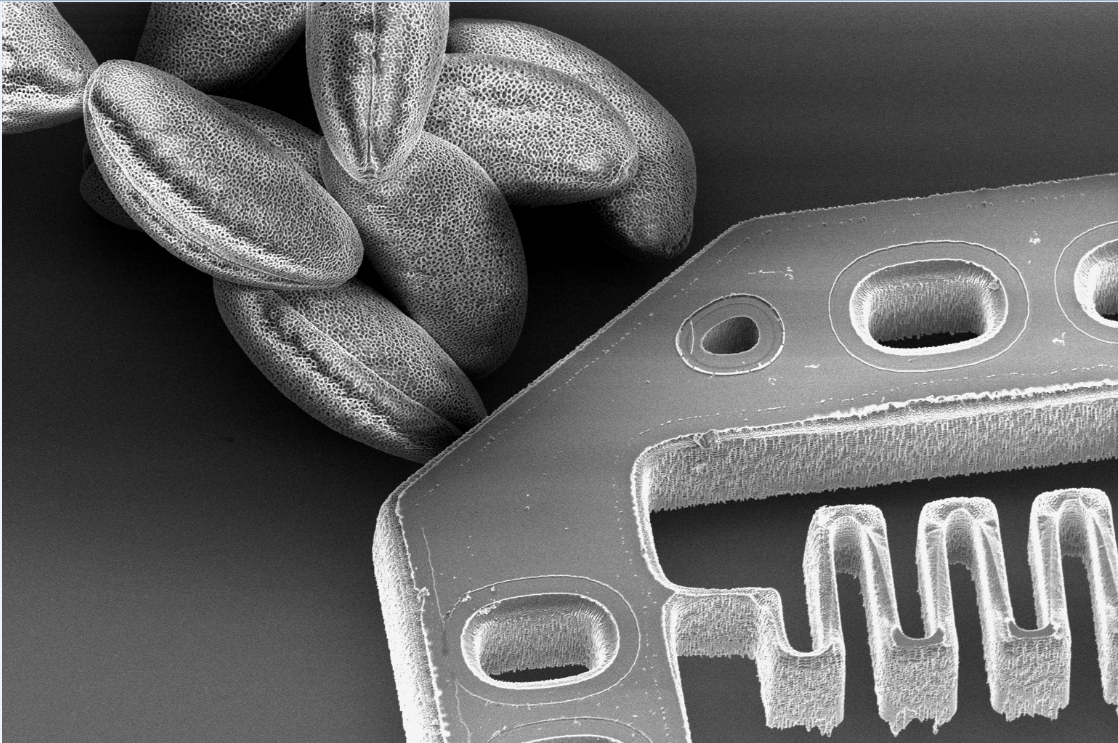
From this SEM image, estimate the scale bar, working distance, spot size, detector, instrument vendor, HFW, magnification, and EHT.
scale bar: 40000 nm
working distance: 13 mm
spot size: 6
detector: ETD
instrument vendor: Thermo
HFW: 195 µm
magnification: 0.65 K X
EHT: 4 kV